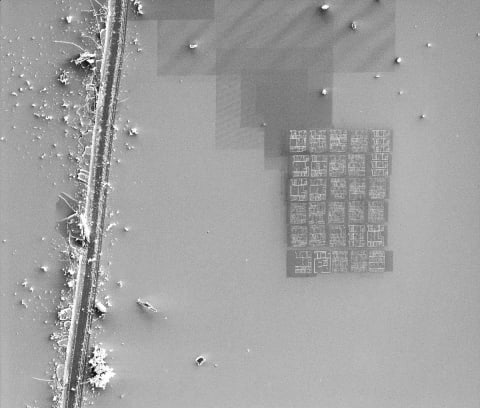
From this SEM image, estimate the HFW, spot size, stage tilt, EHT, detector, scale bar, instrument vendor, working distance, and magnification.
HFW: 304 µm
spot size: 3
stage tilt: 0°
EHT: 5 kV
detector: SED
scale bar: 50000 nm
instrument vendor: FEI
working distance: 5.01 mm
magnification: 0.5 K X